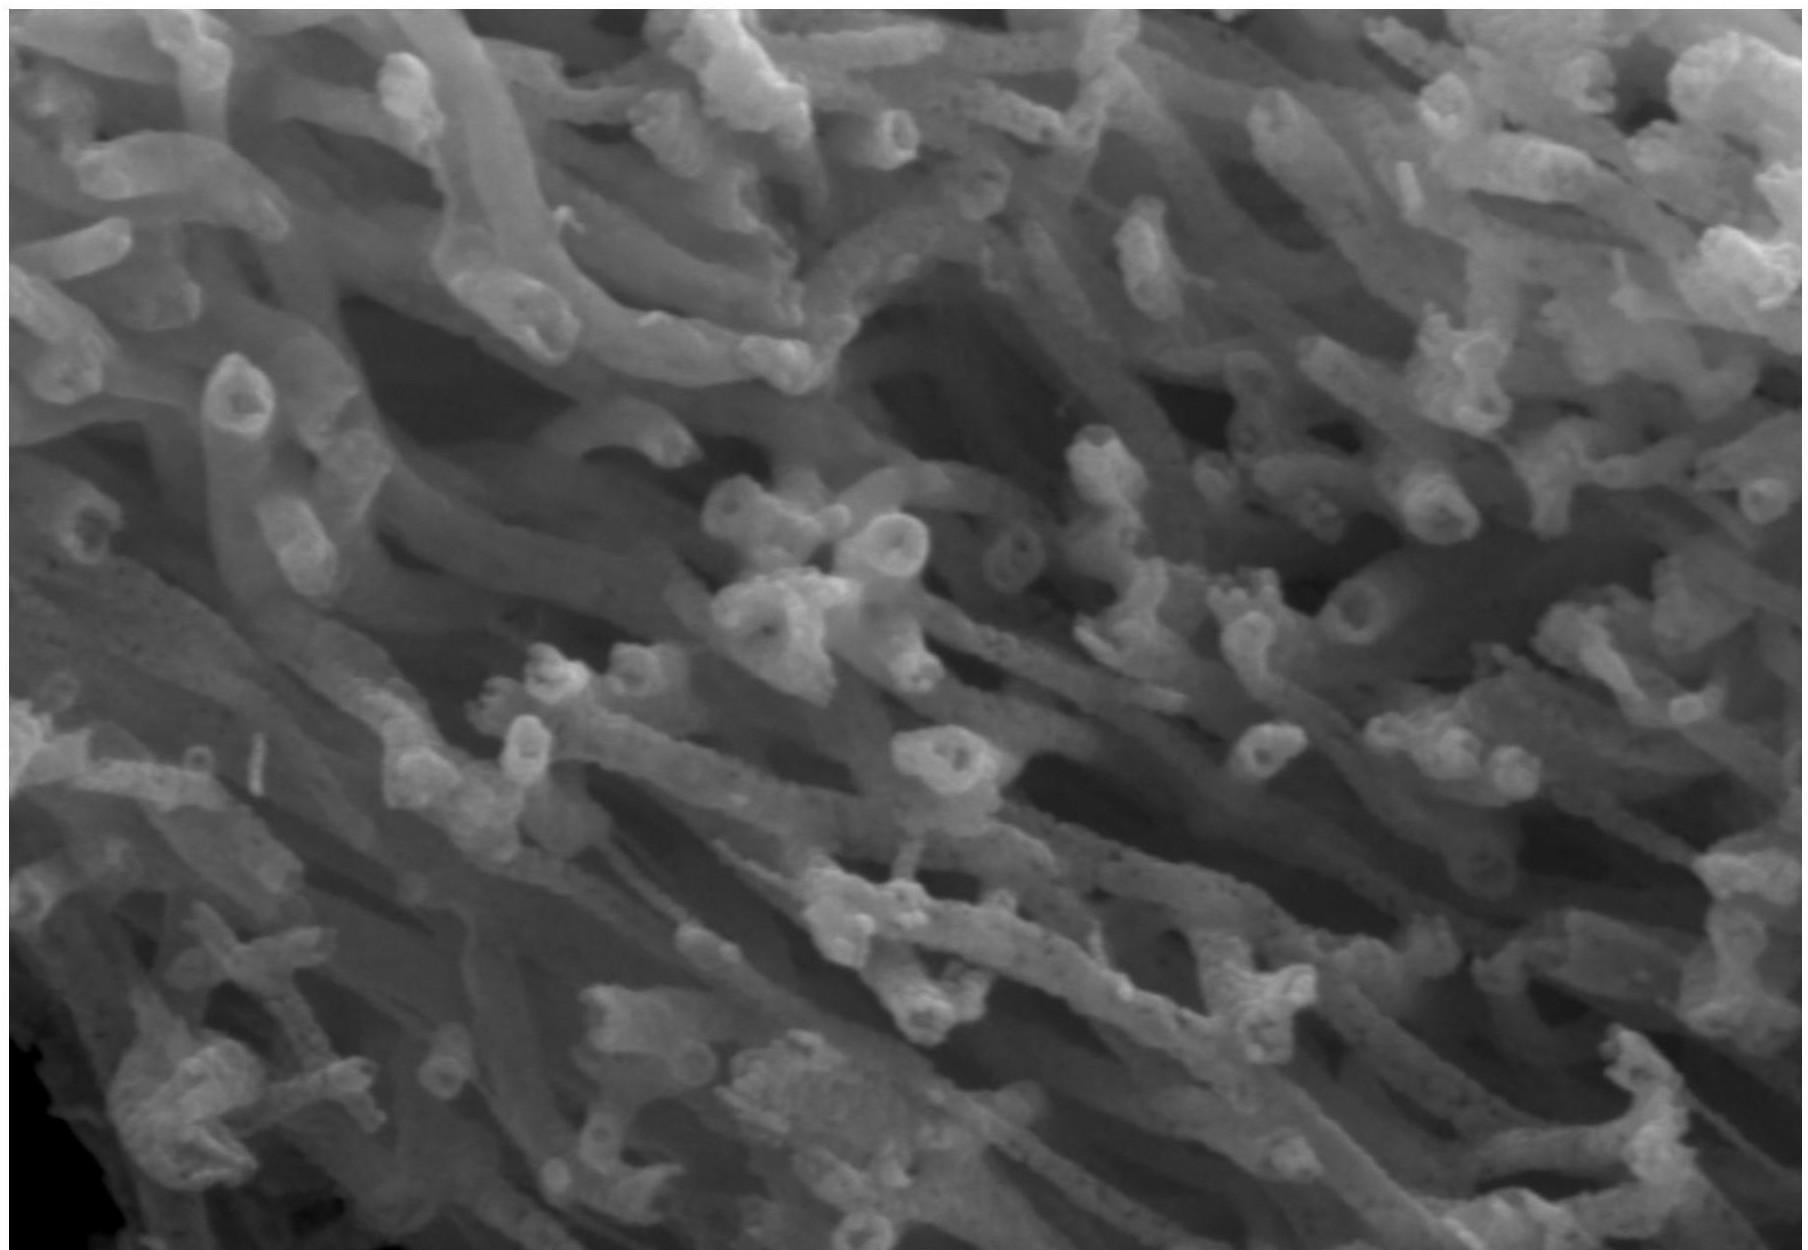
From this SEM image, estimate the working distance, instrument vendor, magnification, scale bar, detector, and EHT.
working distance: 16 mm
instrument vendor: Hitachi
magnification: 20 K X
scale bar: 2000 nm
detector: SE(M)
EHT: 15 kV